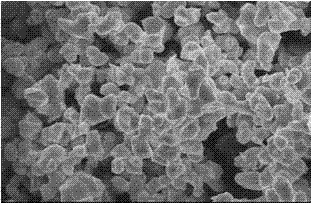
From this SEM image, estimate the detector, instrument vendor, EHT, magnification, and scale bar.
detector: SEI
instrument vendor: JEOL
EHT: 15 kV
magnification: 3 K X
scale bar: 5000 nm